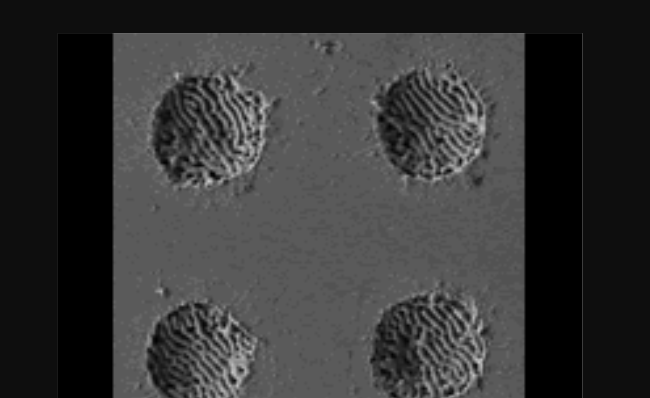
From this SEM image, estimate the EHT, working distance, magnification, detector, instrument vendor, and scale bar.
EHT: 2 kV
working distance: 5.7 mm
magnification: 2 K X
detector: LEI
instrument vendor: JEOL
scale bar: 10000 nm